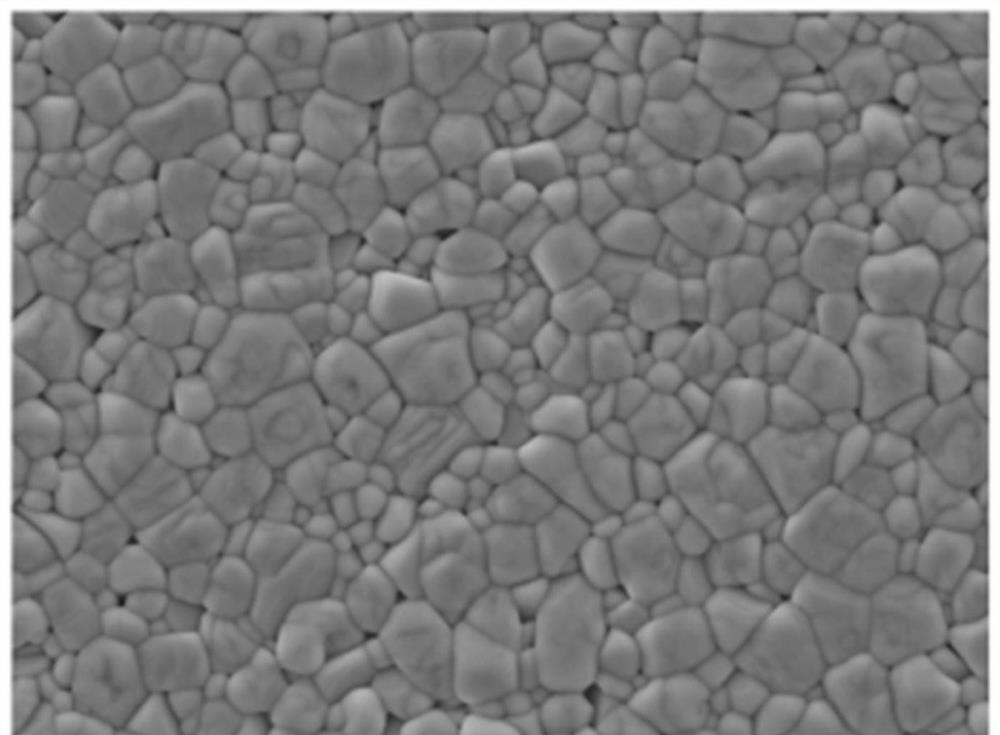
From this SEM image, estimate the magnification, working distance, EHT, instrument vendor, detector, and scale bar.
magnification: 5 K X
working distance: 8.4 mm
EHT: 10 kV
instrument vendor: JEOL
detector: LED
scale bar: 1000 nm